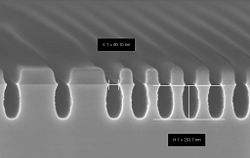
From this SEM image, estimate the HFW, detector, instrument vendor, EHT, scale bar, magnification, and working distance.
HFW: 1.921 µm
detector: InLens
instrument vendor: Zeiss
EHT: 2 kV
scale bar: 200 nm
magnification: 200 K X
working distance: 3.3 mm